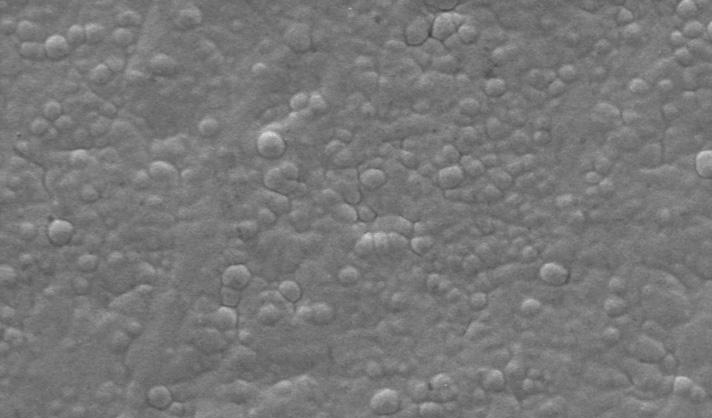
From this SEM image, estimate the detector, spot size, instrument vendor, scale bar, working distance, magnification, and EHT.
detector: SE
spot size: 5.8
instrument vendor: FEI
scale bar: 20000 nm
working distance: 10 mm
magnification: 2 K X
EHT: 15 kV